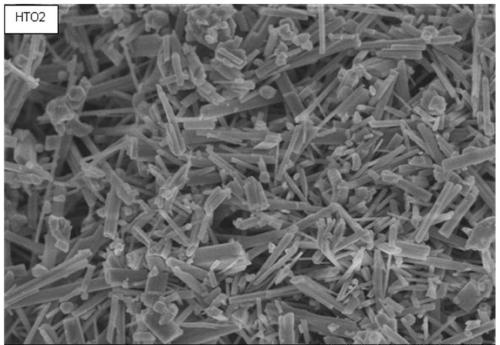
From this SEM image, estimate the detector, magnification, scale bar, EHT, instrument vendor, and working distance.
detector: SE(M)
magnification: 20 K X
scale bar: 2000 nm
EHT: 3 kV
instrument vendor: Hitachi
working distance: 8 mm